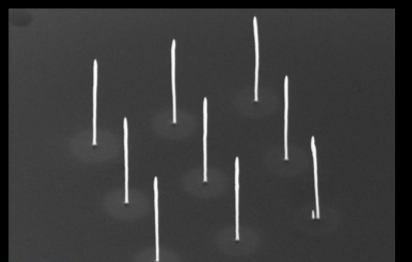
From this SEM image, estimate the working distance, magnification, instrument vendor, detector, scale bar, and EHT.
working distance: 15.8 mm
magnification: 26.72 K X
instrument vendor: Zeiss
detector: SE2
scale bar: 200 nm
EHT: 20 kV